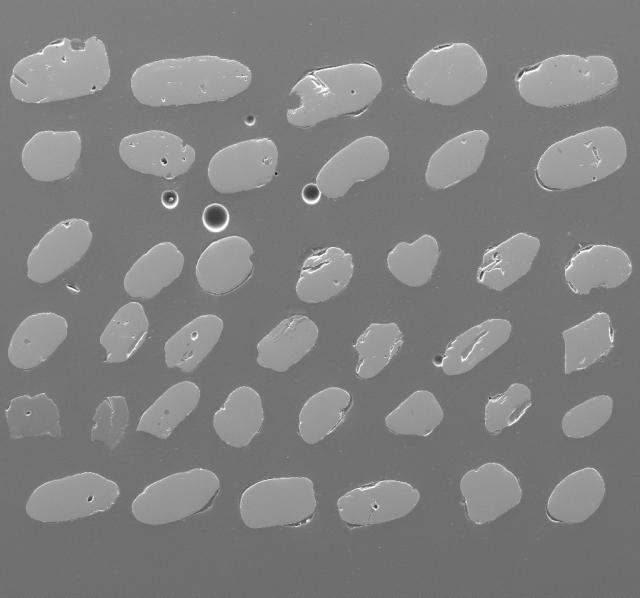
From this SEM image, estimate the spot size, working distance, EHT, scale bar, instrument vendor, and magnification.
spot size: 6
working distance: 18.2 mm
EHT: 15 kV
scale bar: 500000 nm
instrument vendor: FEI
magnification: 0.116 K X